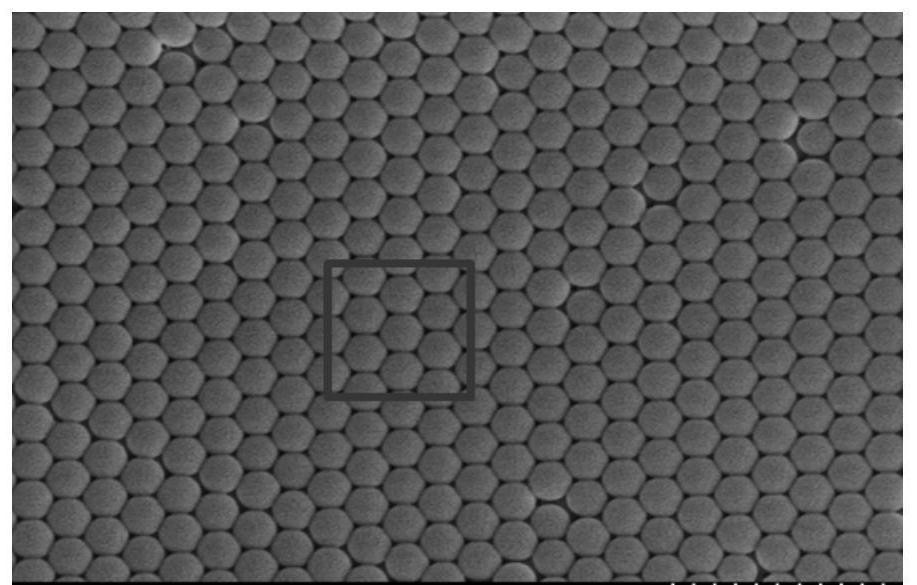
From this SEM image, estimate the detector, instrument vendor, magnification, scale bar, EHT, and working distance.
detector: SE(M)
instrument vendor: Hitachi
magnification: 30 K X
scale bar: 1000 nm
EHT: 10 kV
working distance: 13.1 mm